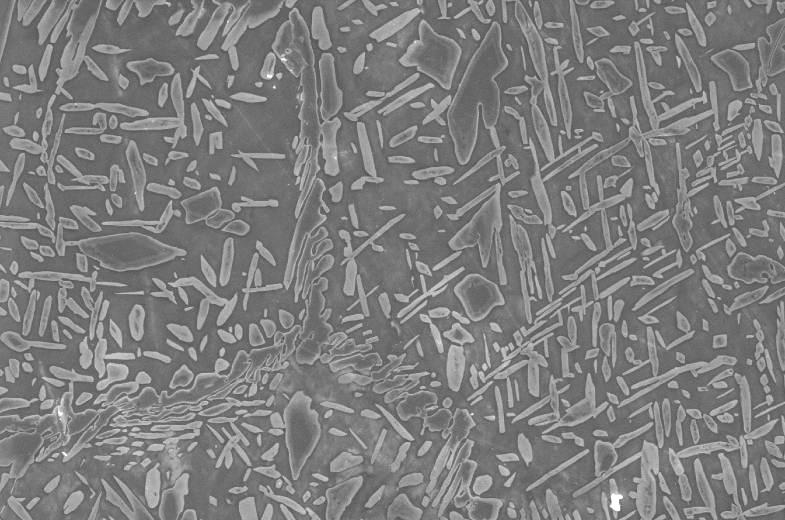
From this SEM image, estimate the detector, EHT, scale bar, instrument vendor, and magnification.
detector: SEI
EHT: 20 kV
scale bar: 10000 nm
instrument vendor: JEOL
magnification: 1 K X